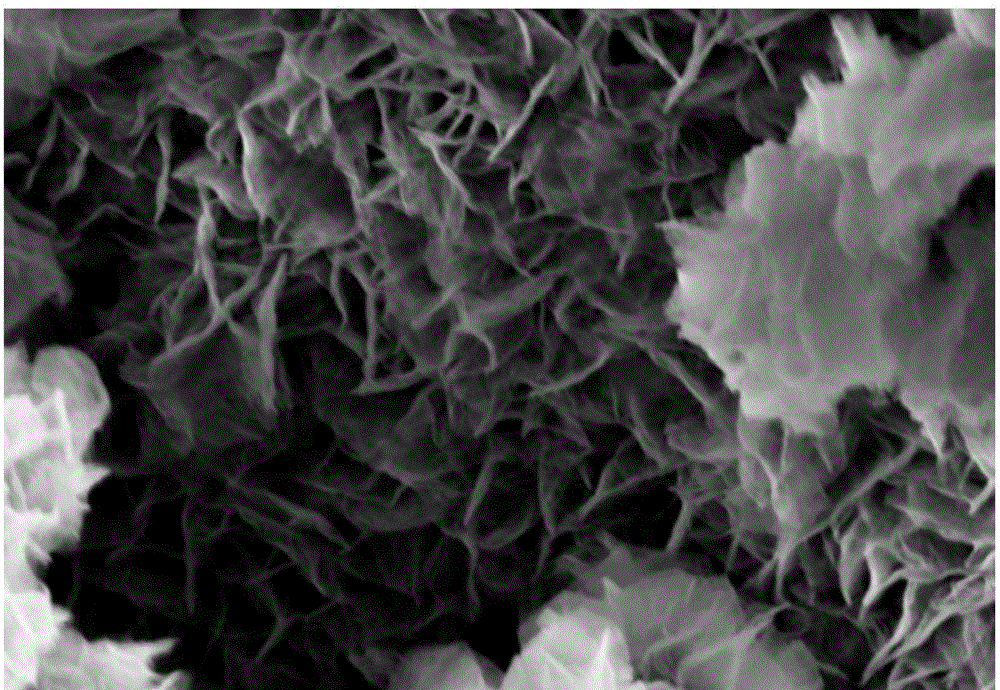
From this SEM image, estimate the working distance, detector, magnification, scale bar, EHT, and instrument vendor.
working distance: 7.2 mm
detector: InLens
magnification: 30 K X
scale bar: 100 nm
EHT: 5 kV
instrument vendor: Zeiss